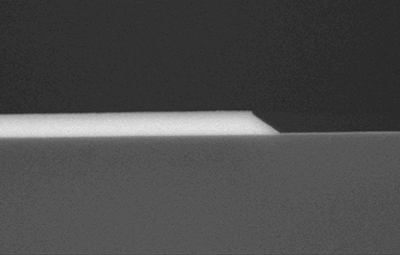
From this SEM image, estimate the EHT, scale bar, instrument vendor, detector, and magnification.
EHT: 10 kV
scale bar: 1000 nm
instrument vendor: Hitachi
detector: YAGBSE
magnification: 30 K X